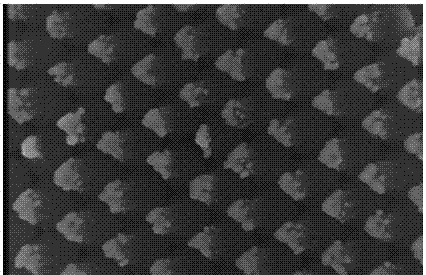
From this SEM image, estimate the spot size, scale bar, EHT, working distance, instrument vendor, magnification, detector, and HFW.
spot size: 3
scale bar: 300 nm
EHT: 17 kV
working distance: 4.9 mm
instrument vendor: FEI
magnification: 140 K X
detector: TLD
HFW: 1.48 µm